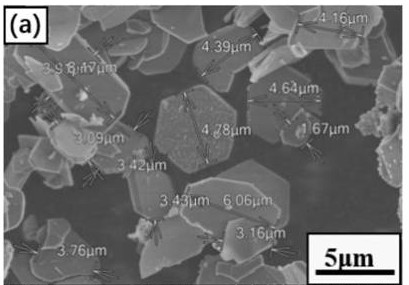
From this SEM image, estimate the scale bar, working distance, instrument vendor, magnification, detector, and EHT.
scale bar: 10000 nm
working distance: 6.1 mm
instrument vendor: Hitachi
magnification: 5 K X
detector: SE(L)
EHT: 5 kV